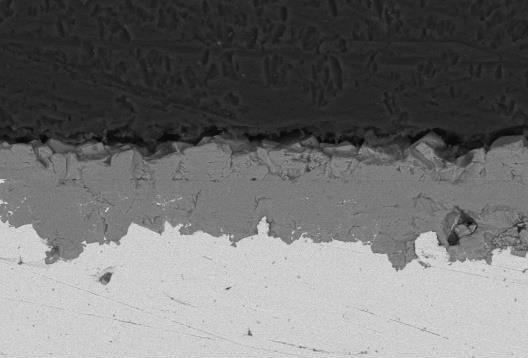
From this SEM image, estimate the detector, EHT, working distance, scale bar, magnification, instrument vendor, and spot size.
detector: BEC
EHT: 15 kV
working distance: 11 mm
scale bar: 50000 nm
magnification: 0.5 K X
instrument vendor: JEOL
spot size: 40